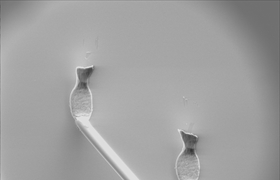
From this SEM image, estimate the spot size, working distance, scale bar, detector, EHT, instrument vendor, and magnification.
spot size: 5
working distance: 10.2 mm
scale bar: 100000 nm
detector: GSE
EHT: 20 kV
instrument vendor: FEI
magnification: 0.2 K X